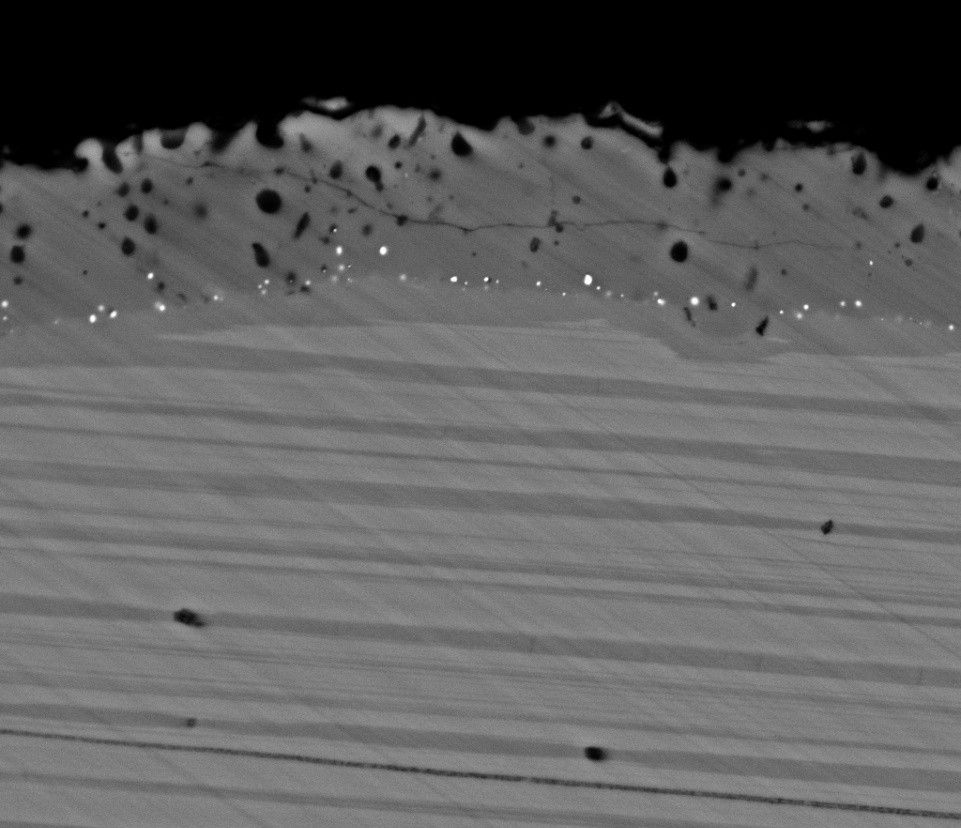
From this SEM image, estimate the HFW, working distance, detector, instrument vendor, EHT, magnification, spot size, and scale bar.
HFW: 37.3 µm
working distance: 6 mm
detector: BSED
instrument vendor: FEI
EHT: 18 kV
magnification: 8 K X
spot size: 4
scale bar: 10000 nm